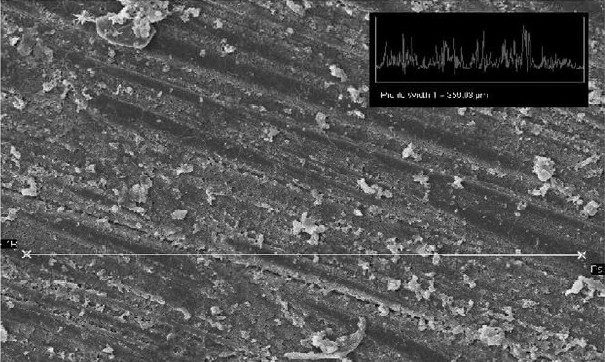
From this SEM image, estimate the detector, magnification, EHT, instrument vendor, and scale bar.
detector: SE1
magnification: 0.3 K X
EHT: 20 kV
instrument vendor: Zeiss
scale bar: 100000 nm